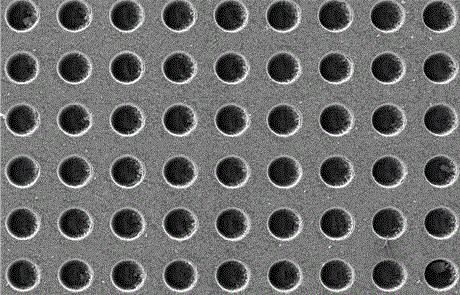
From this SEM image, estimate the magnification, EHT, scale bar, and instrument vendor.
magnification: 0.05 K X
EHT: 20 kV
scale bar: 500000 nm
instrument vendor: JEOL